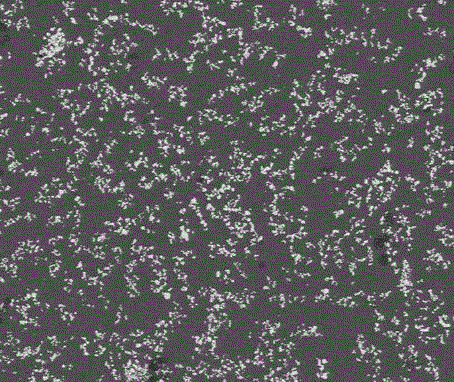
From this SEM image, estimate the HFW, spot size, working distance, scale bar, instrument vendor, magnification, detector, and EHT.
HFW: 746 µm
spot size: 2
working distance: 8.9 mm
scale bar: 200000 nm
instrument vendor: FEI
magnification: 0.2 K X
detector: BSED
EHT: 15 kV